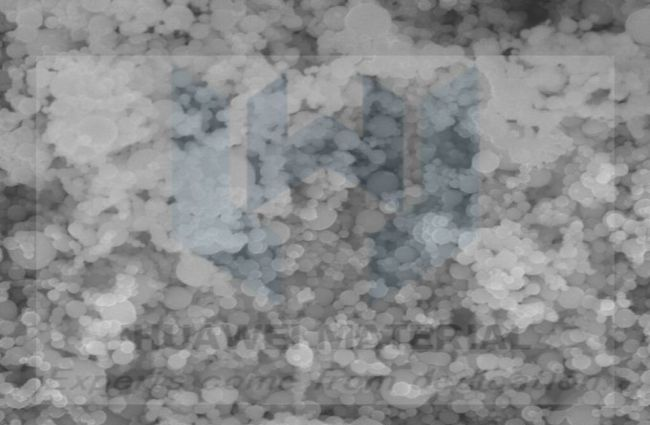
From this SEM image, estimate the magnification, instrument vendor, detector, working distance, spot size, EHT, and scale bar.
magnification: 100 K X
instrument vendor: FEI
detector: TLD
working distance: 5.3 mm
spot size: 3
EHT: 15 kV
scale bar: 500 nm